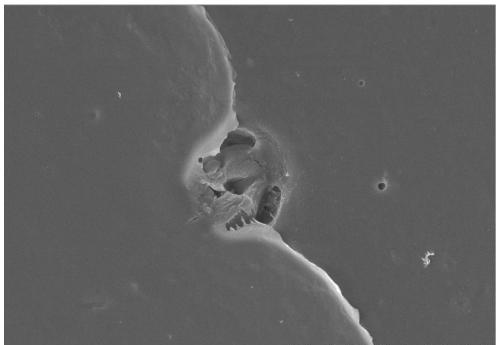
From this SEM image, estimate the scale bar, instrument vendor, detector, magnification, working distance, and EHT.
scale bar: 10000 nm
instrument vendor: Hitachi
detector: SE(U)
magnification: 5 K X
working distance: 7.4 mm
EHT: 5 kV